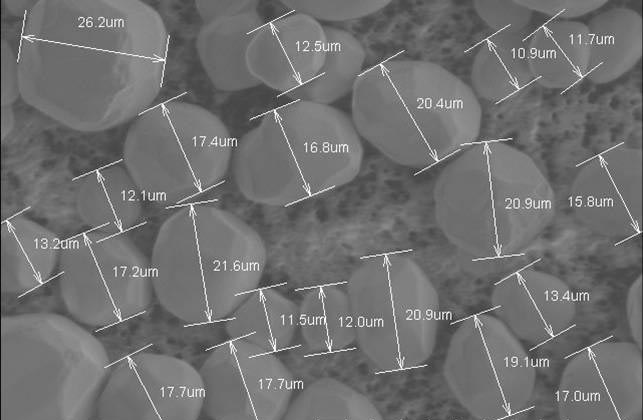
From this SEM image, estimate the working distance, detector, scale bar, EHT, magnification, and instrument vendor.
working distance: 10.1 mm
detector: SE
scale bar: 50000 nm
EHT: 3 kV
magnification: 1.1 K X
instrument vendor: Hitachi